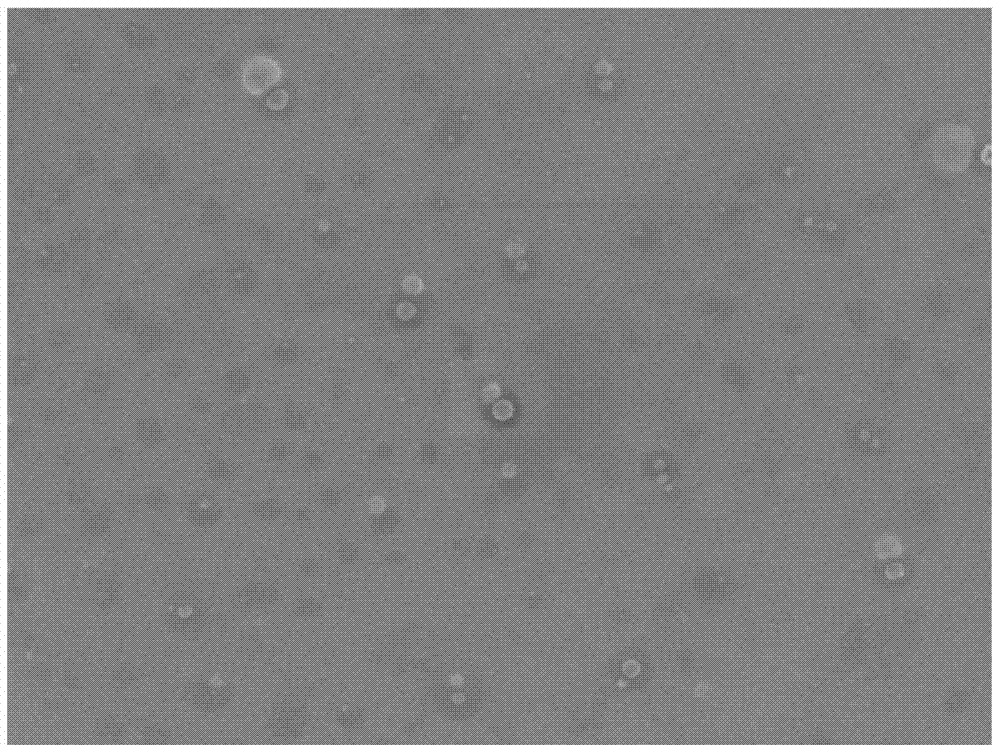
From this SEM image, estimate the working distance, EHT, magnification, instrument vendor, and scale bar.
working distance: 6.7 mm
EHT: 5 kV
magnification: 3 K X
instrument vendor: JEOL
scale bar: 1000 nm